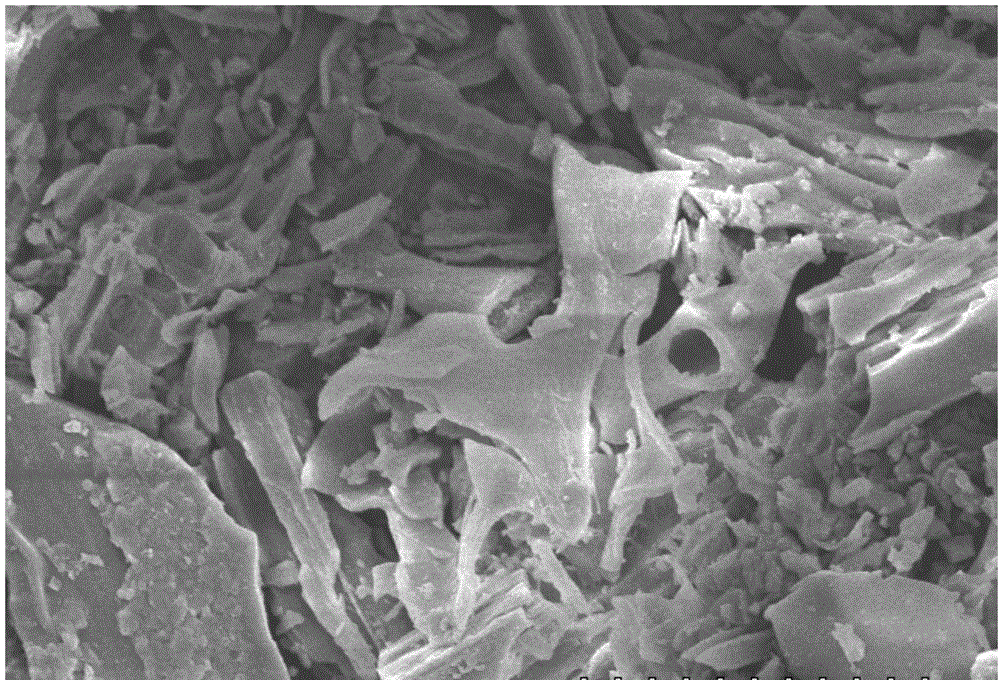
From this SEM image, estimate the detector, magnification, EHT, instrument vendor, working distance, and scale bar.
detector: SE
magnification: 1.5 K X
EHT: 10 kV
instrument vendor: Hitachi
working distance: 15.2 mm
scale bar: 30000 nm